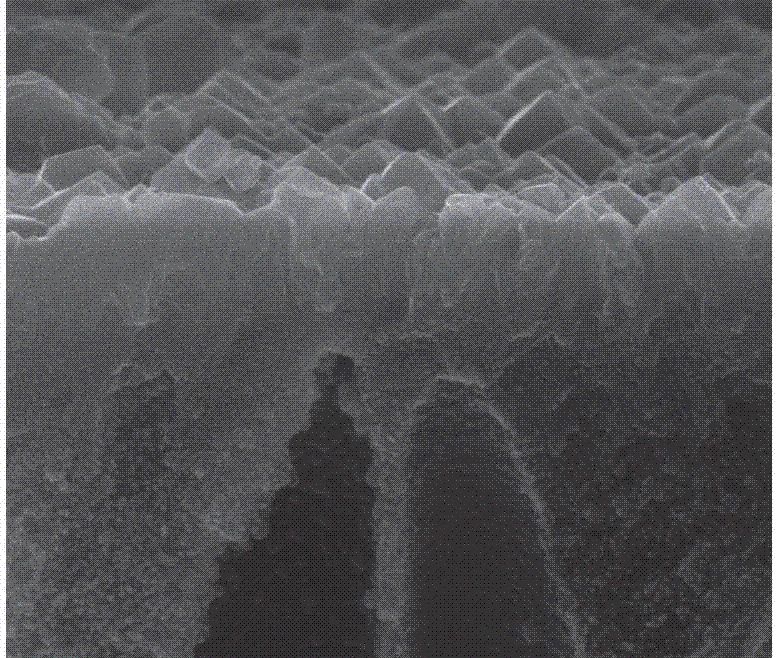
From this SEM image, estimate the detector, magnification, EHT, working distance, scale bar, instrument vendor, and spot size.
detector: ETD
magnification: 20 K X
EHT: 20 kV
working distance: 10 mm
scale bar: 5000 nm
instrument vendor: FEI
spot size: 3